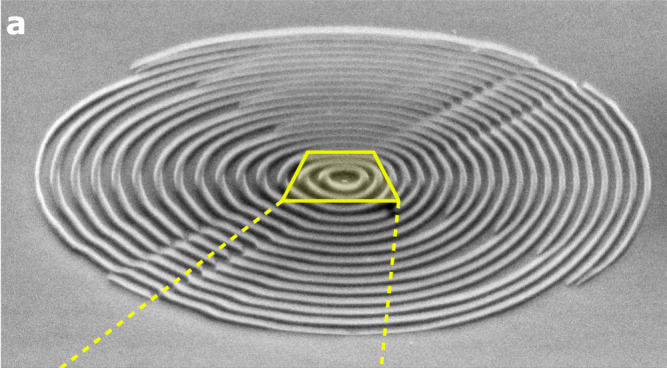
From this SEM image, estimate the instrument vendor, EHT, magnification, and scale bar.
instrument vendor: JEOL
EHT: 30 kV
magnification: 7 K X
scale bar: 2000 nm